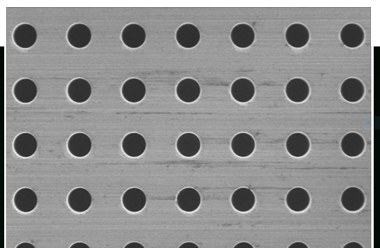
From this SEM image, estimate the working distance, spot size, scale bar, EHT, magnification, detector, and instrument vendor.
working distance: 11 mm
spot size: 50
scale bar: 100000 nm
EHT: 1 kV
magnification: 0.2 K X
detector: SEI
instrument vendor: JEOL